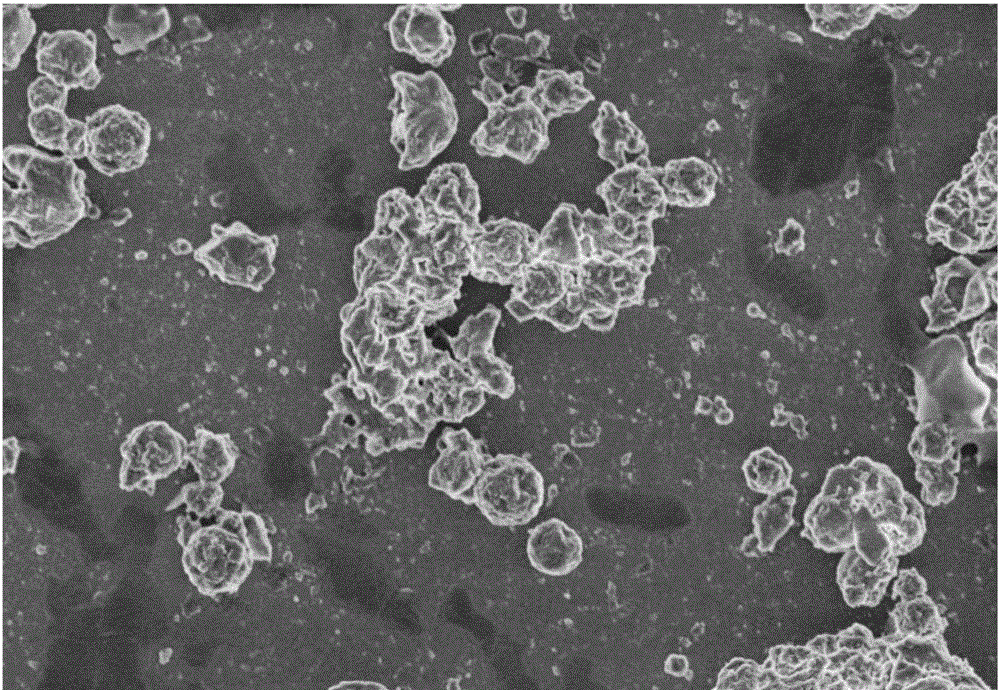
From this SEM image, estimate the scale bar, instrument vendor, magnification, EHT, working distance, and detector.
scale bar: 10000 nm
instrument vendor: Hitachi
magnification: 4 K X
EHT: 3 kV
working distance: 8.4 mm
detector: SE(U)